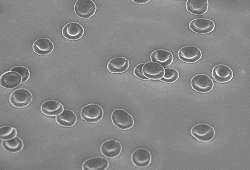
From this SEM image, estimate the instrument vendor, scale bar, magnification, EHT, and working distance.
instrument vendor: Hitachi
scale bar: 30000 nm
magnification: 1.5 K X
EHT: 3 kV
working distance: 8.8 mm